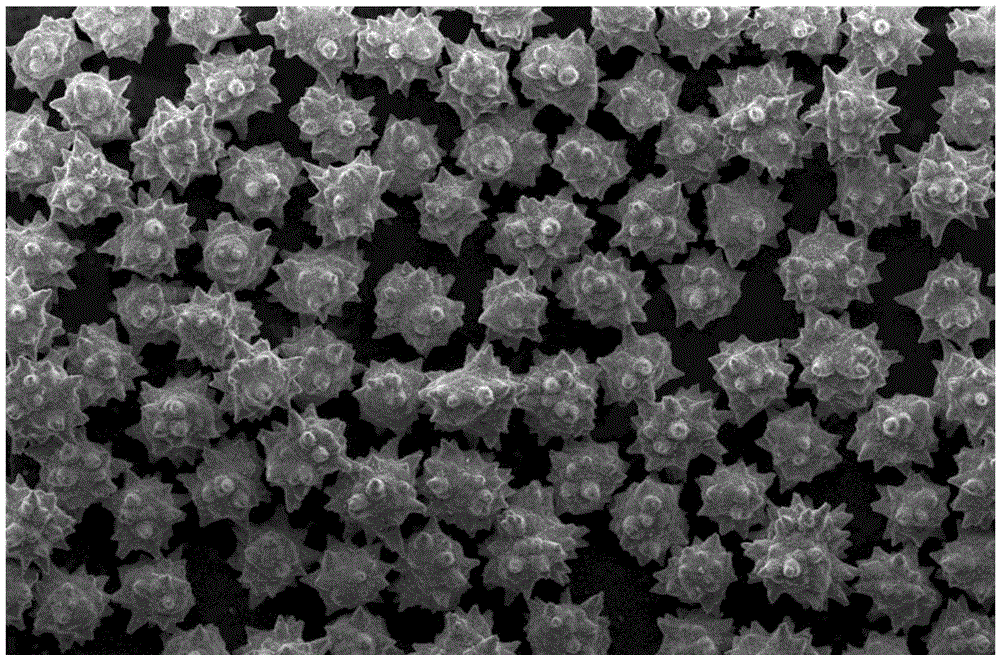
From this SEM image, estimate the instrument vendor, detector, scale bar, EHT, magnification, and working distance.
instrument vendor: FEI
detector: ETD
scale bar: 100000 nm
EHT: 5 kV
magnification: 1 K X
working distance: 9.8 mm